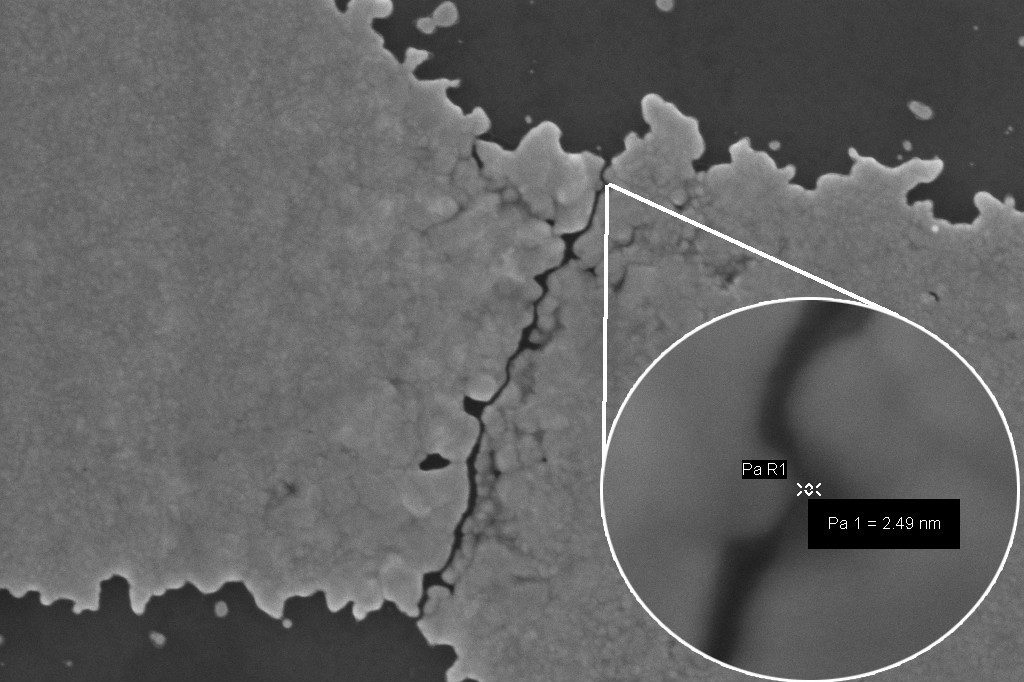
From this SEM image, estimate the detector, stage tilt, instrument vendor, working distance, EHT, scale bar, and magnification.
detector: InLens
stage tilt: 0°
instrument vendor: Zeiss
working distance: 3 mm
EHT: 5 kV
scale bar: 200 nm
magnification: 100 K X